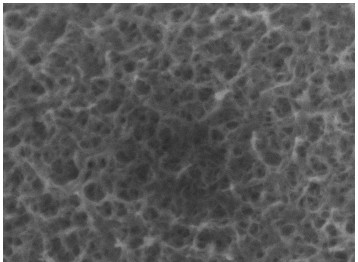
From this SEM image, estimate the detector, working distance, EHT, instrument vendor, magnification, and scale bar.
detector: SEI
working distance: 9.1 mm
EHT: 10 kV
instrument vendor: JEOL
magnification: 30 K X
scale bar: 100 nm